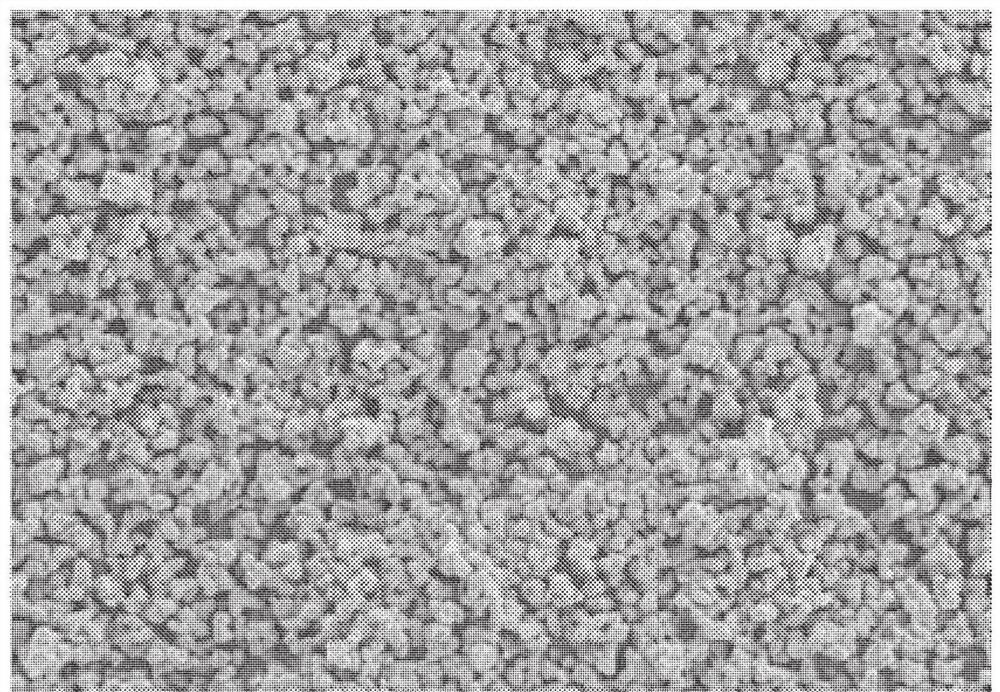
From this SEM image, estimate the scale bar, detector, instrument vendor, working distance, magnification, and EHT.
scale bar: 100000 nm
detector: SE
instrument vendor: Hitachi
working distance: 4.6 mm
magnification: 0.5 K X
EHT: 10 kV